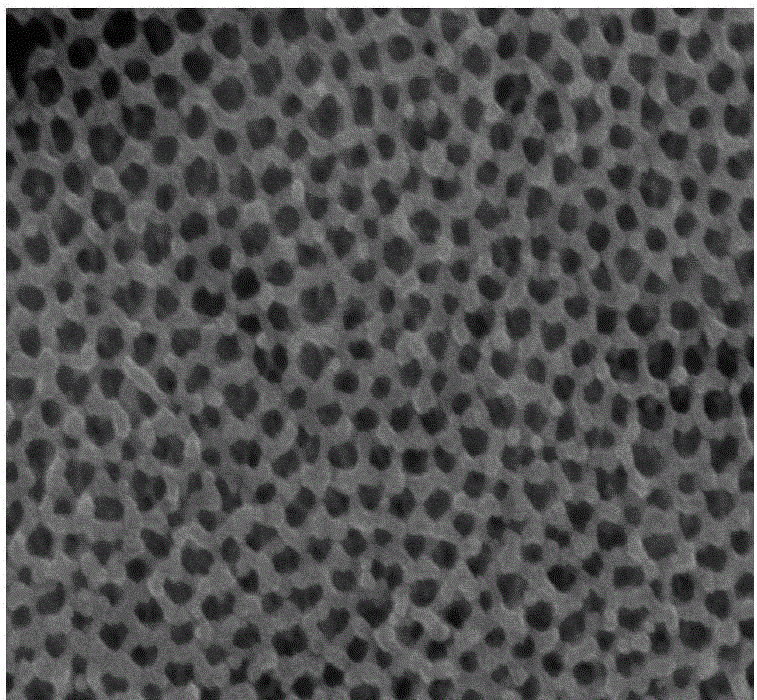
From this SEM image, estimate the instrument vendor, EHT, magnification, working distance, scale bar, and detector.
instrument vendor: Hitachi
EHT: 10 kV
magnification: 110 K X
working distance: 9.1 mm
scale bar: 500 nm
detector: SE(M)